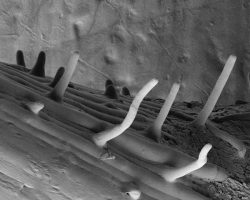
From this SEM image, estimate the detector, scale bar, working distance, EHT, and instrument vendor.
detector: SE2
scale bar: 100000 nm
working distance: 18.3 mm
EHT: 3 kV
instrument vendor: Zeiss